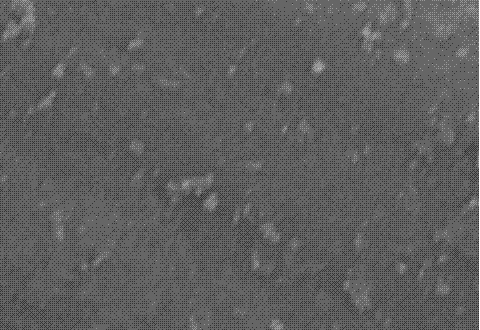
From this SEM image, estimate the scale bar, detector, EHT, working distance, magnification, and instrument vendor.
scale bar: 3000 nm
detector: SE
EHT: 15 kV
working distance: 14.4 mm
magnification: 17 K X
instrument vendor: Hitachi